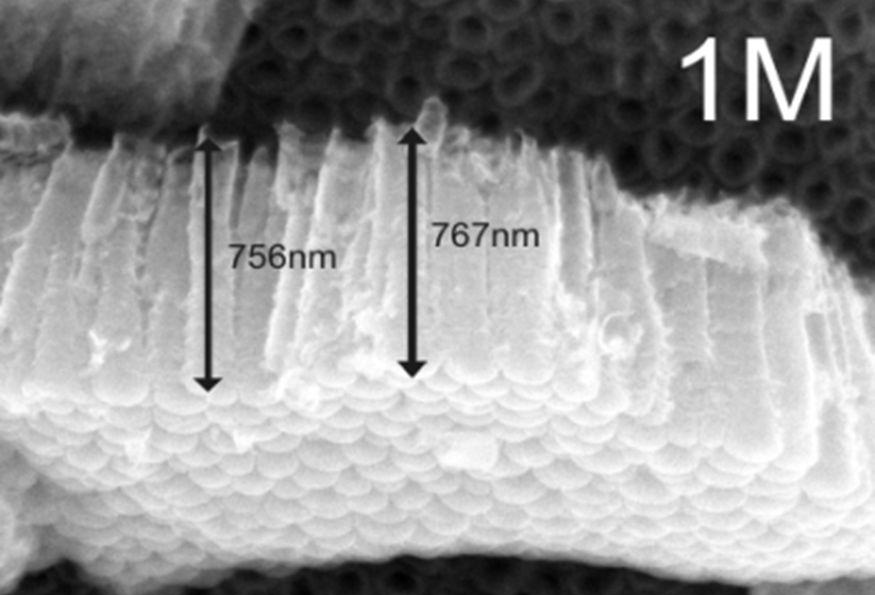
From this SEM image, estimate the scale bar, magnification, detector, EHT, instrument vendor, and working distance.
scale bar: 100 nm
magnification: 50 K X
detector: SEI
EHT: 12 kV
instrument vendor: JEOL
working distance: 8.3 mm